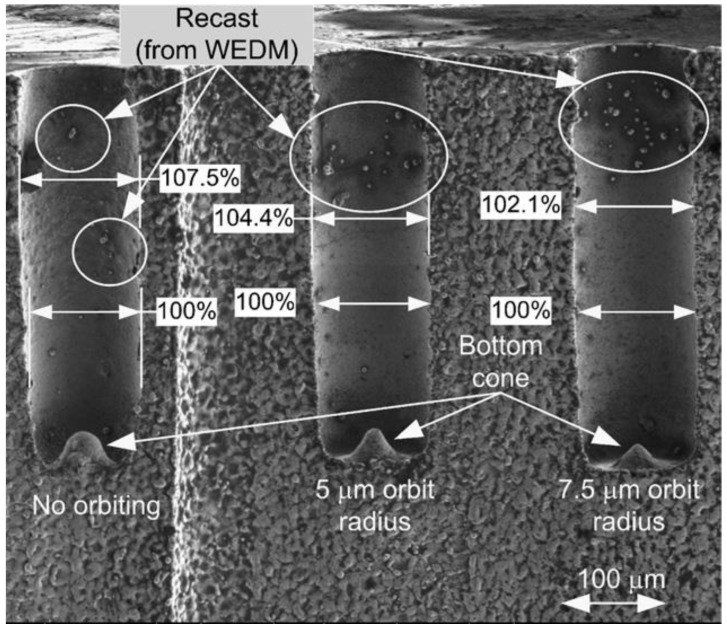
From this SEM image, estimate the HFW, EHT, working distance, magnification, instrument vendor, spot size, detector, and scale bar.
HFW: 686 µm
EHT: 1 kV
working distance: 4.8 mm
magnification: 0.35 K X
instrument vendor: FEI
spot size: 4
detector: ETD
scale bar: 100000 nm